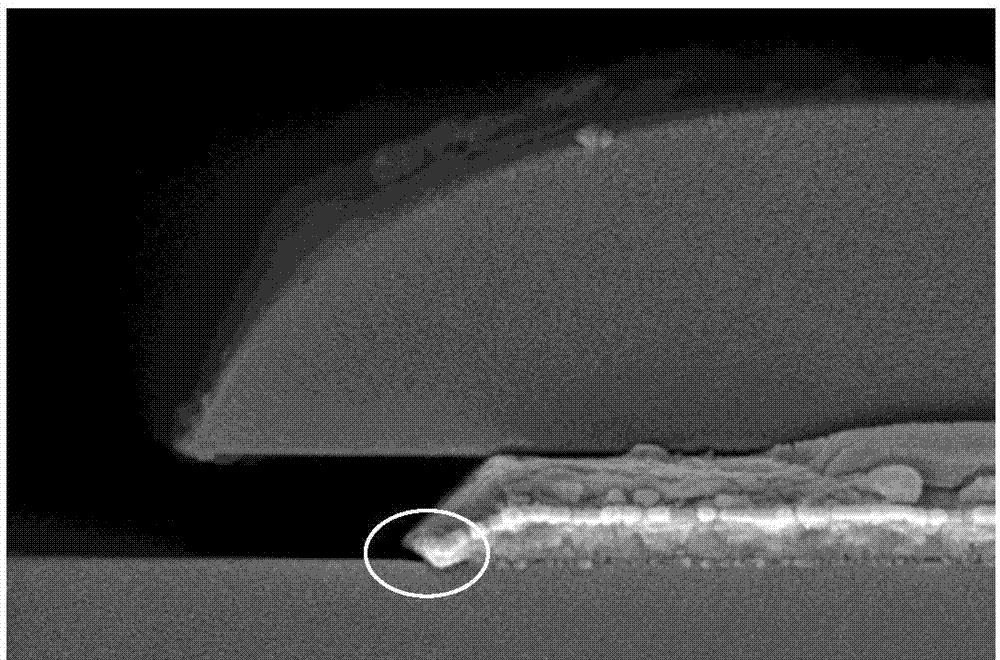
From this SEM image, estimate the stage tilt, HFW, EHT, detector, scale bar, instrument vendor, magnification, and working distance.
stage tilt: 0°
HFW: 4.14 µm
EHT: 5 kV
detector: T2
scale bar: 1000 nm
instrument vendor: FEI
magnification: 100 K X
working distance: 7 mm